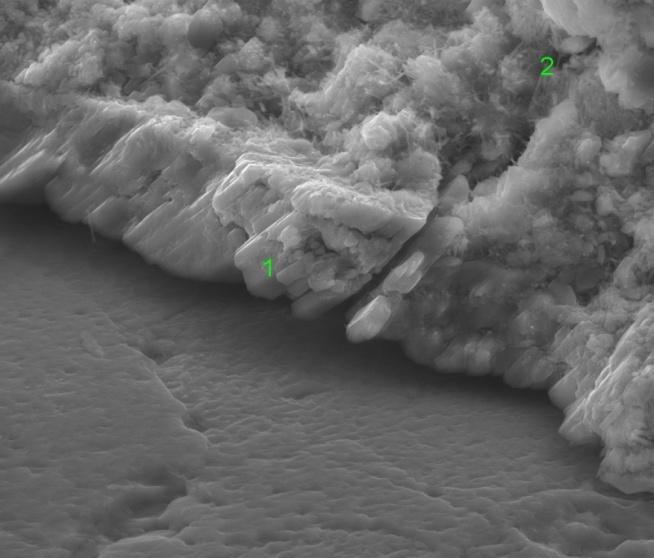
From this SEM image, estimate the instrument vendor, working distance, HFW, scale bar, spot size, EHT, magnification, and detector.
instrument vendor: FEI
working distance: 6.9 mm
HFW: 74.6 µm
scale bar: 30000 nm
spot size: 4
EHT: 18 kV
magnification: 4 K X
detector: LVD